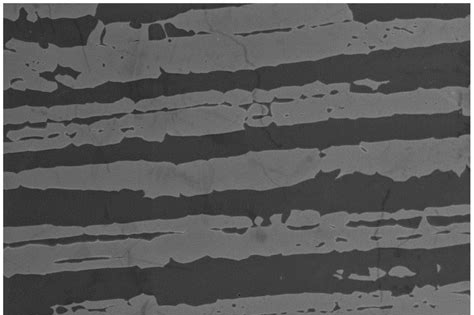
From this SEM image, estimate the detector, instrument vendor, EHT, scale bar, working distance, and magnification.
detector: SEI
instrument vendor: JEOL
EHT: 20 kV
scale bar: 50000 nm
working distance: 10 mm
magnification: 0.5 K X